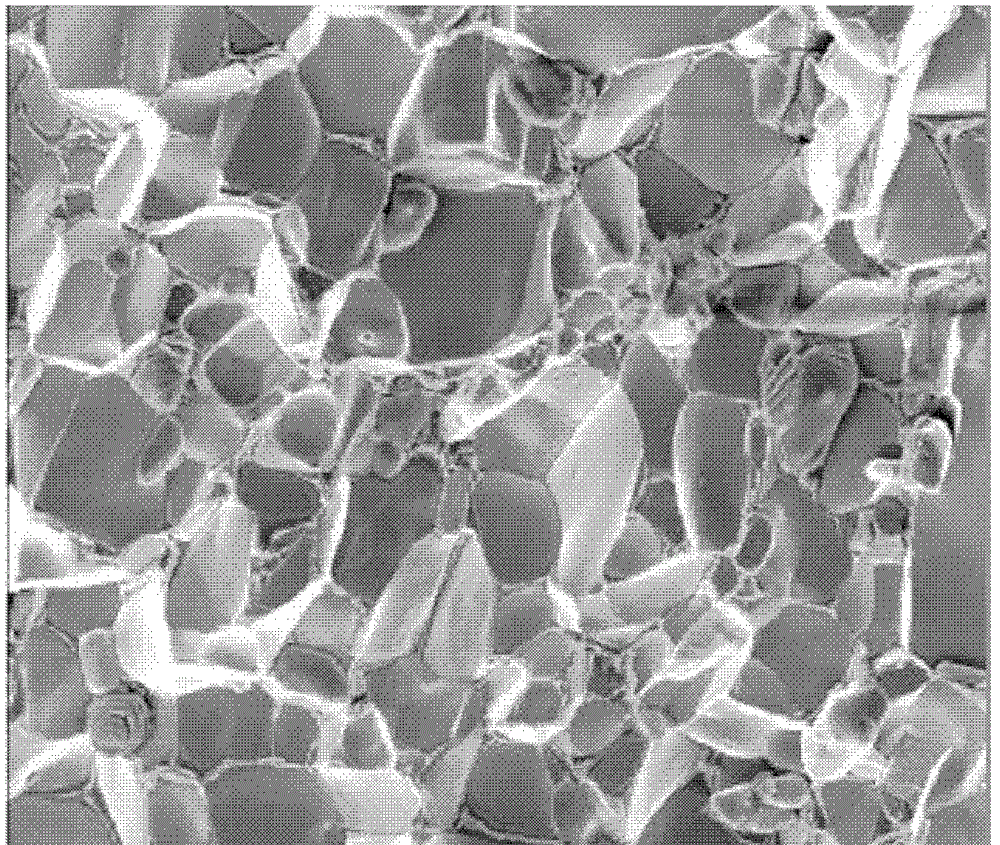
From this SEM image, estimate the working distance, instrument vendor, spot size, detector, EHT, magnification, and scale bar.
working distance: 11 mm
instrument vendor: FEI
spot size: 3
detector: ETD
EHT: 5 kV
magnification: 10 K X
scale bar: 5000 nm